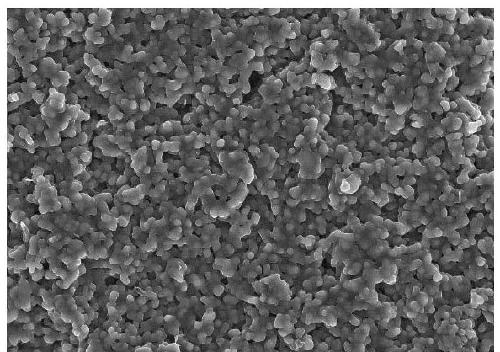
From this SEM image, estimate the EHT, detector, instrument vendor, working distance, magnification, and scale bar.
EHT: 10 kV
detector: SE(UL)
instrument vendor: Hitachi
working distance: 8.9 mm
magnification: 5 K X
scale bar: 10000 nm